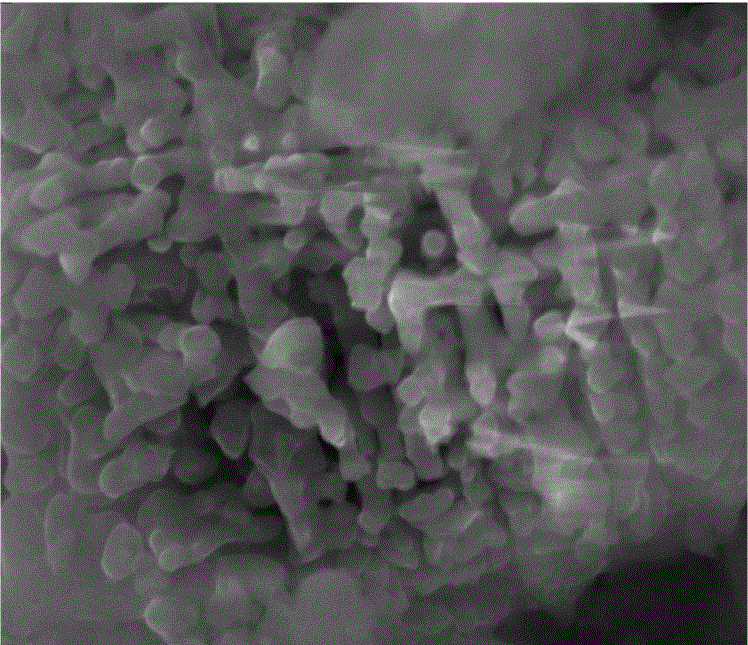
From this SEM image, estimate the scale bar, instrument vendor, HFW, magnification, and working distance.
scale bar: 5000 nm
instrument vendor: FEI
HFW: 13.52 µm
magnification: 10 K X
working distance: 11.9 mm